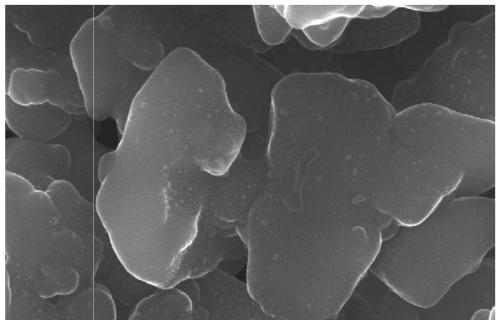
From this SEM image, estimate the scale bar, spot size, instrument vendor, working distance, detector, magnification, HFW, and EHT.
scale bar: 3000 nm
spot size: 3.5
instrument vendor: FEI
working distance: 9.8 mm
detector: ETD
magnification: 50 K X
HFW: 8.29 µm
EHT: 20 kV